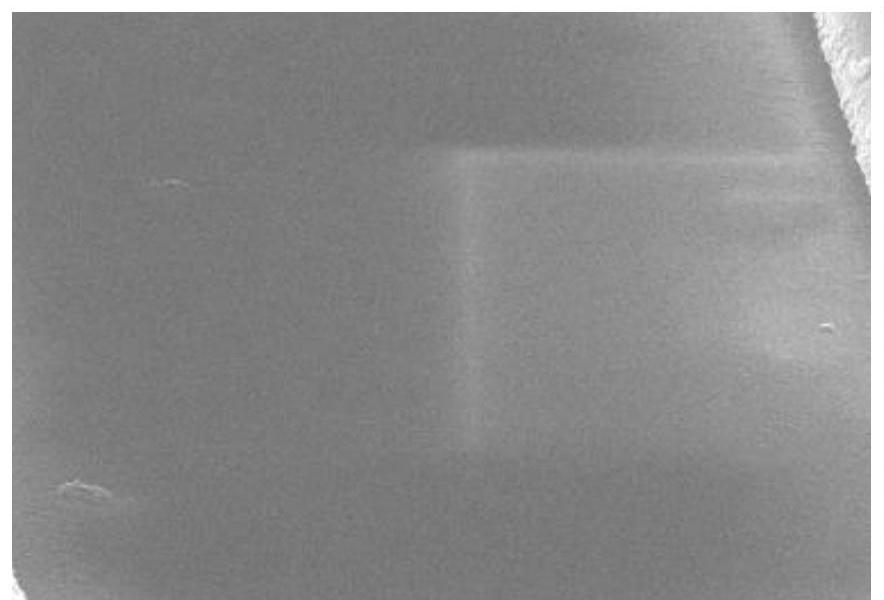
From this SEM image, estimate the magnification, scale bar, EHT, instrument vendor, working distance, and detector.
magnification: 20 K X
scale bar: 2000 nm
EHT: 5 kV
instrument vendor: Hitachi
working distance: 12.4 mm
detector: SE(UL)